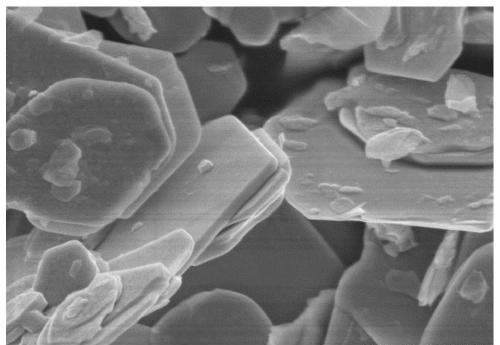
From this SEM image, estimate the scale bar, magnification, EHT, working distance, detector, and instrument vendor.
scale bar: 1000 nm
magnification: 30 K X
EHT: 5 kV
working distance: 10.4 mm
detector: SE(U)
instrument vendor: Hitachi